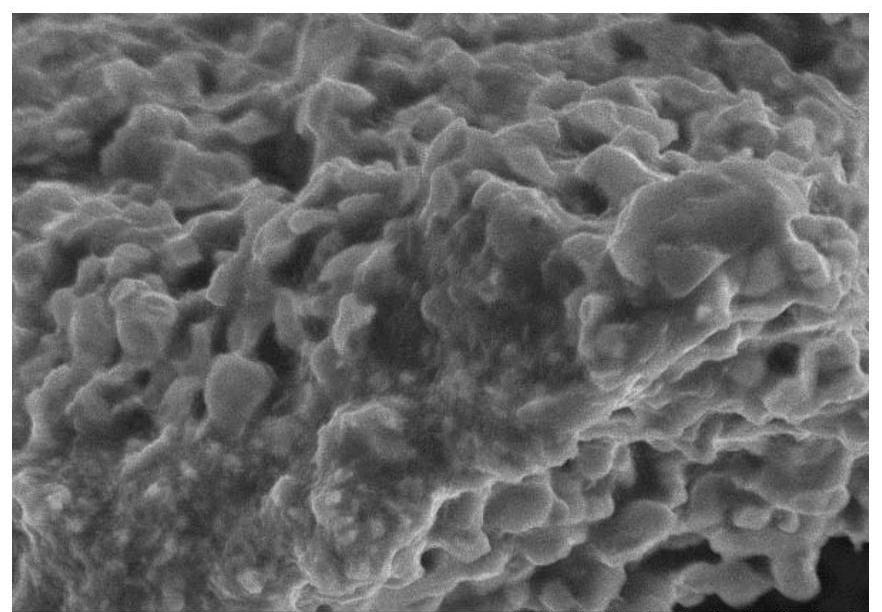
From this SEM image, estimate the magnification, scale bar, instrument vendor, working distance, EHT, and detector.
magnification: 2.5 K X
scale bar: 20000 nm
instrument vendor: Hitachi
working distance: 10 mm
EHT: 15 kV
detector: SE